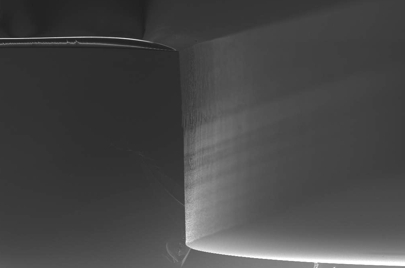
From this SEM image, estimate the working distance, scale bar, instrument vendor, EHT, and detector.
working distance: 4 mm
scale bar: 30000 nm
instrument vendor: Zeiss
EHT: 5 kV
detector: InLens